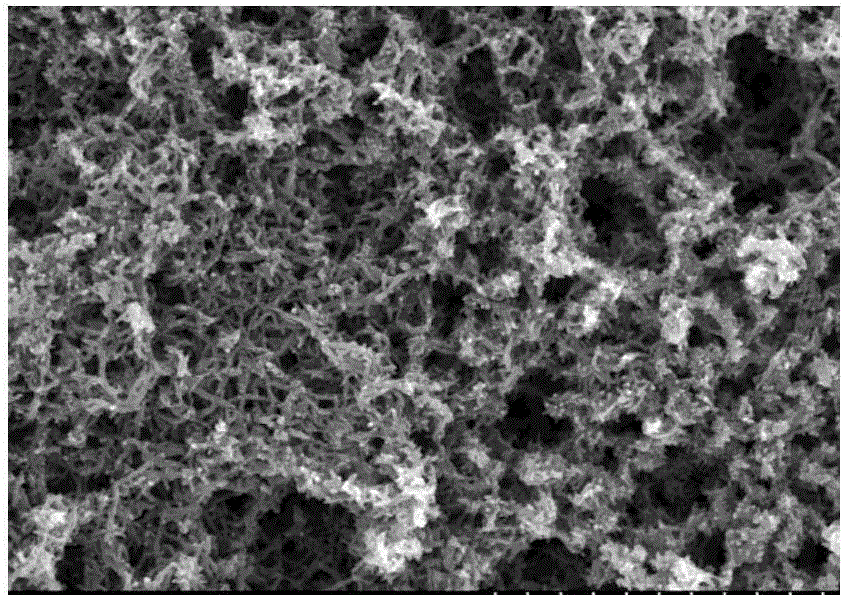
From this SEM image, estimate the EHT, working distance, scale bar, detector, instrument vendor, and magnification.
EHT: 10 kV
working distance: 8.7 mm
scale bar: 10000 nm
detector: SE(M)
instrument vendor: Hitachi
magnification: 5 K X